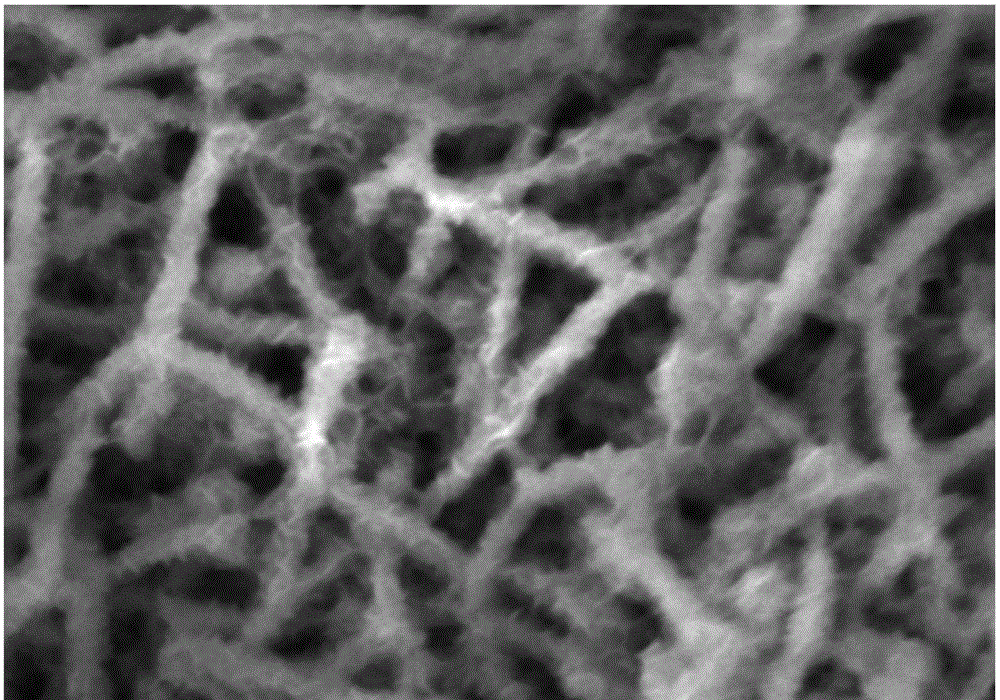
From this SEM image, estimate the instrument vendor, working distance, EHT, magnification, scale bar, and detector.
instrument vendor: JEOL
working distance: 4 mm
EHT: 3 kV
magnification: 100 K X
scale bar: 100 nm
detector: UED